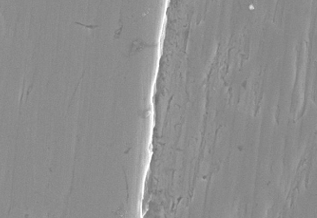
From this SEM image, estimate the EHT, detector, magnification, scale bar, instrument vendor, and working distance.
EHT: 10 kV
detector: SE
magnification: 3 K X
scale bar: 10000 nm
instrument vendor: JEOL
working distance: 15.1 mm